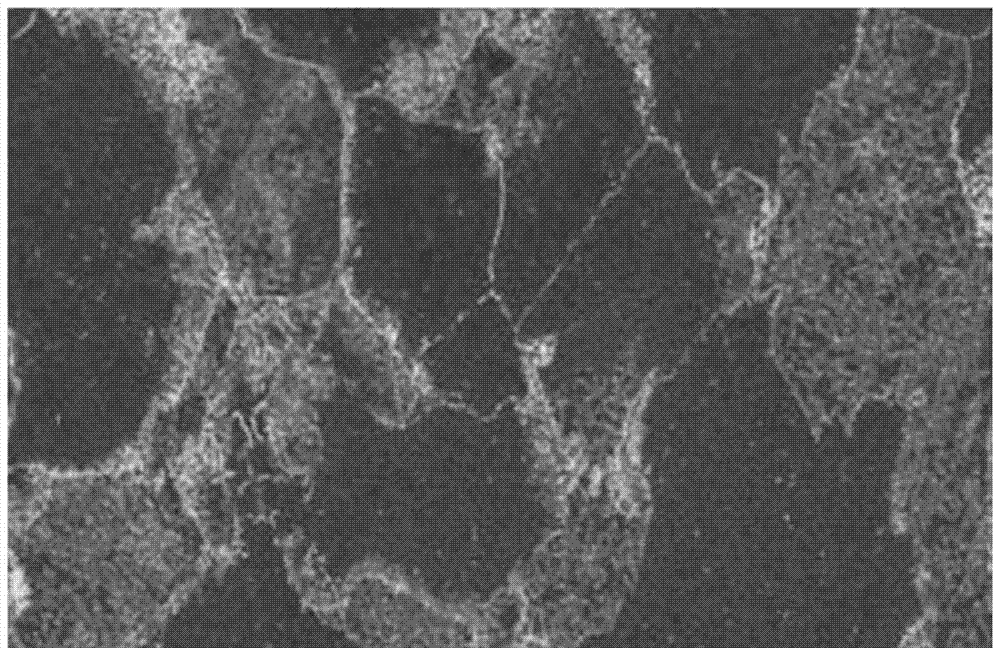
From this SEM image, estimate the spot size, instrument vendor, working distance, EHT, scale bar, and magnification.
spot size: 3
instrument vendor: FEI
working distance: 7.9 mm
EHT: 2 kV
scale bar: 5000 nm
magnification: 8 K X